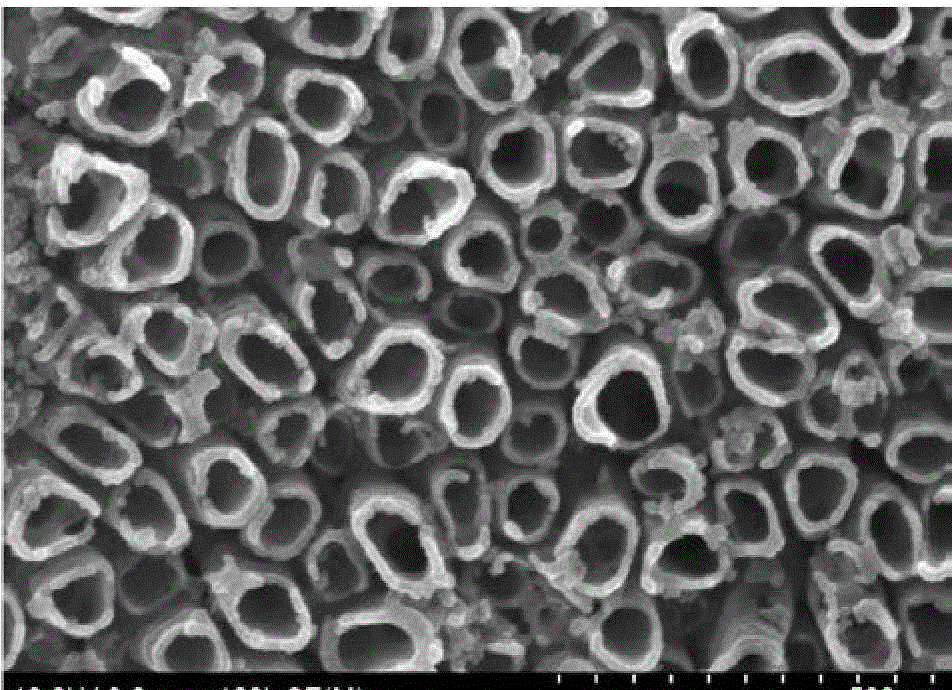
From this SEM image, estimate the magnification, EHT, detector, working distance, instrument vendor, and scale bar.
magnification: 100 K X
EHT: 10 kV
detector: SE(M)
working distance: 8 mm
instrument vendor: Hitachi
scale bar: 500 nm